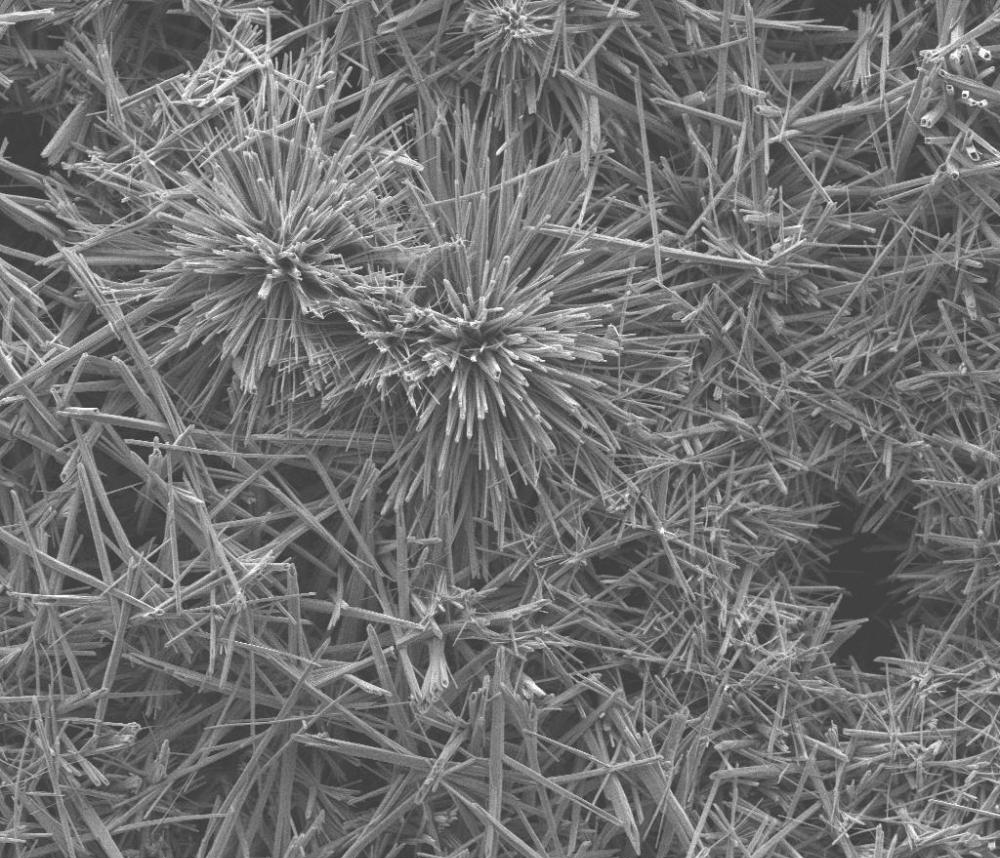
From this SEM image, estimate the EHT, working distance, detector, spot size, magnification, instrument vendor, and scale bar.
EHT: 10 kV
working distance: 6.9 mm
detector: ETD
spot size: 3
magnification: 1.2 K X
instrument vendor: FEI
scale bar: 50000 nm